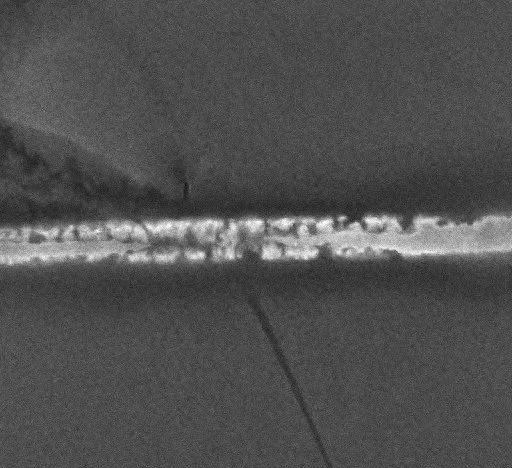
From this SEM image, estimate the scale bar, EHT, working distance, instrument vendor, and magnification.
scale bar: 2000 nm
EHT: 10 kV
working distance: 4 mm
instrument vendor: JEOL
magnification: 10 K X